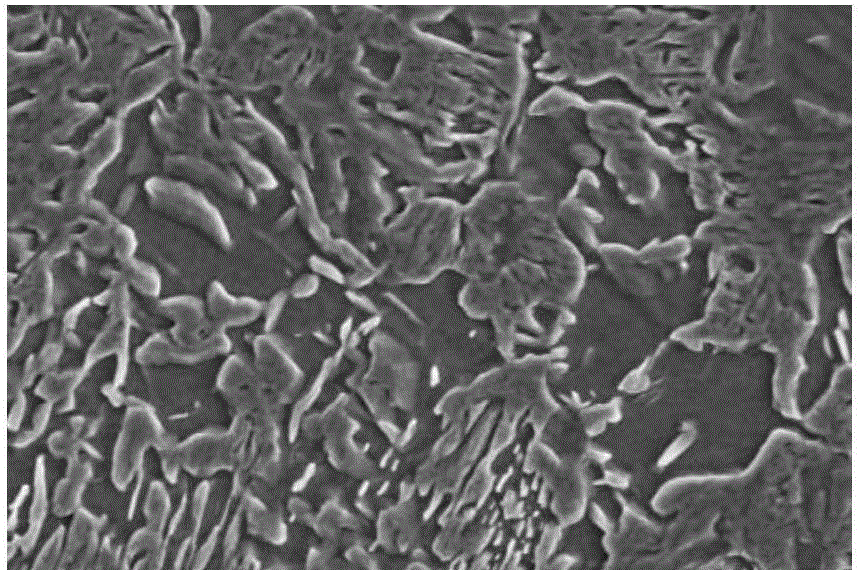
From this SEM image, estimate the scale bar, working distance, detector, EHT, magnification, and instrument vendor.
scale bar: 20000 nm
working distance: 15 mm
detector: SE(M)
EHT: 20 kV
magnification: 2 K X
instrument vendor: Hitachi